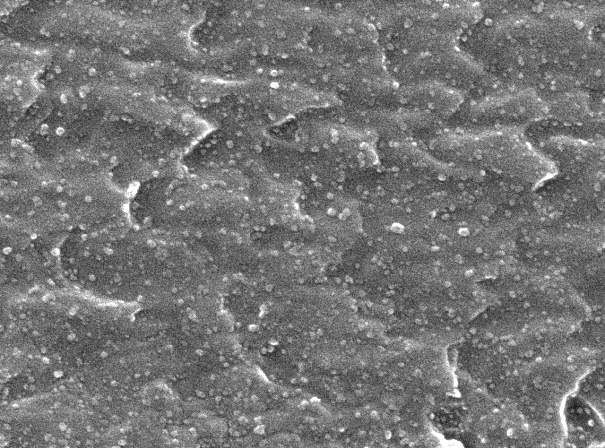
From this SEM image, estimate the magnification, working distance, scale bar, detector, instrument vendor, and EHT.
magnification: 50 K X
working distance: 10 mm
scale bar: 100 nm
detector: SEI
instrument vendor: JEOL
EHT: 15 kV